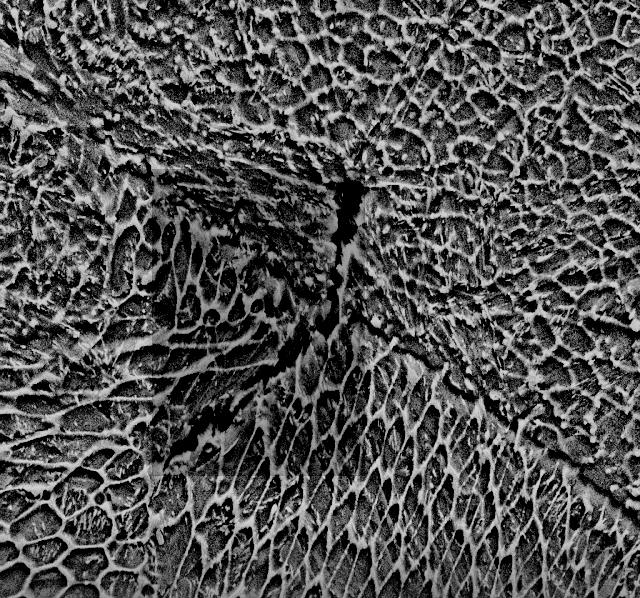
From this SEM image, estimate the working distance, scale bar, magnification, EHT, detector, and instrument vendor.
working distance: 8.3 mm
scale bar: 3000 nm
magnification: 10 K X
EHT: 15 kV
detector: SE2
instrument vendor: Zeiss